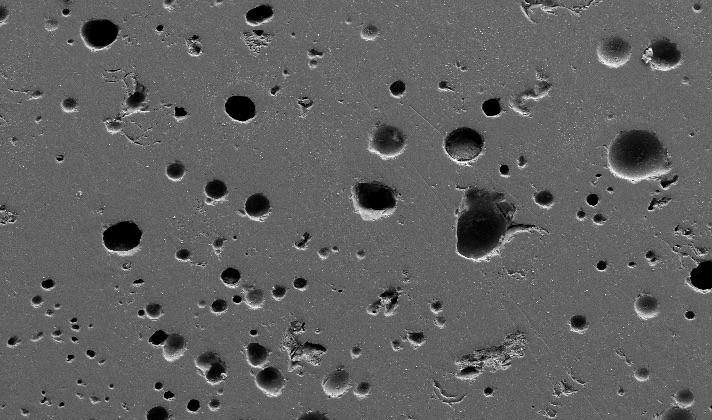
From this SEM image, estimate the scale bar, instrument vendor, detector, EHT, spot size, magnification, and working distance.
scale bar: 100000 nm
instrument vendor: FEI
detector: SE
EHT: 15 kV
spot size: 3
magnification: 0.2 K X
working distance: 5 mm